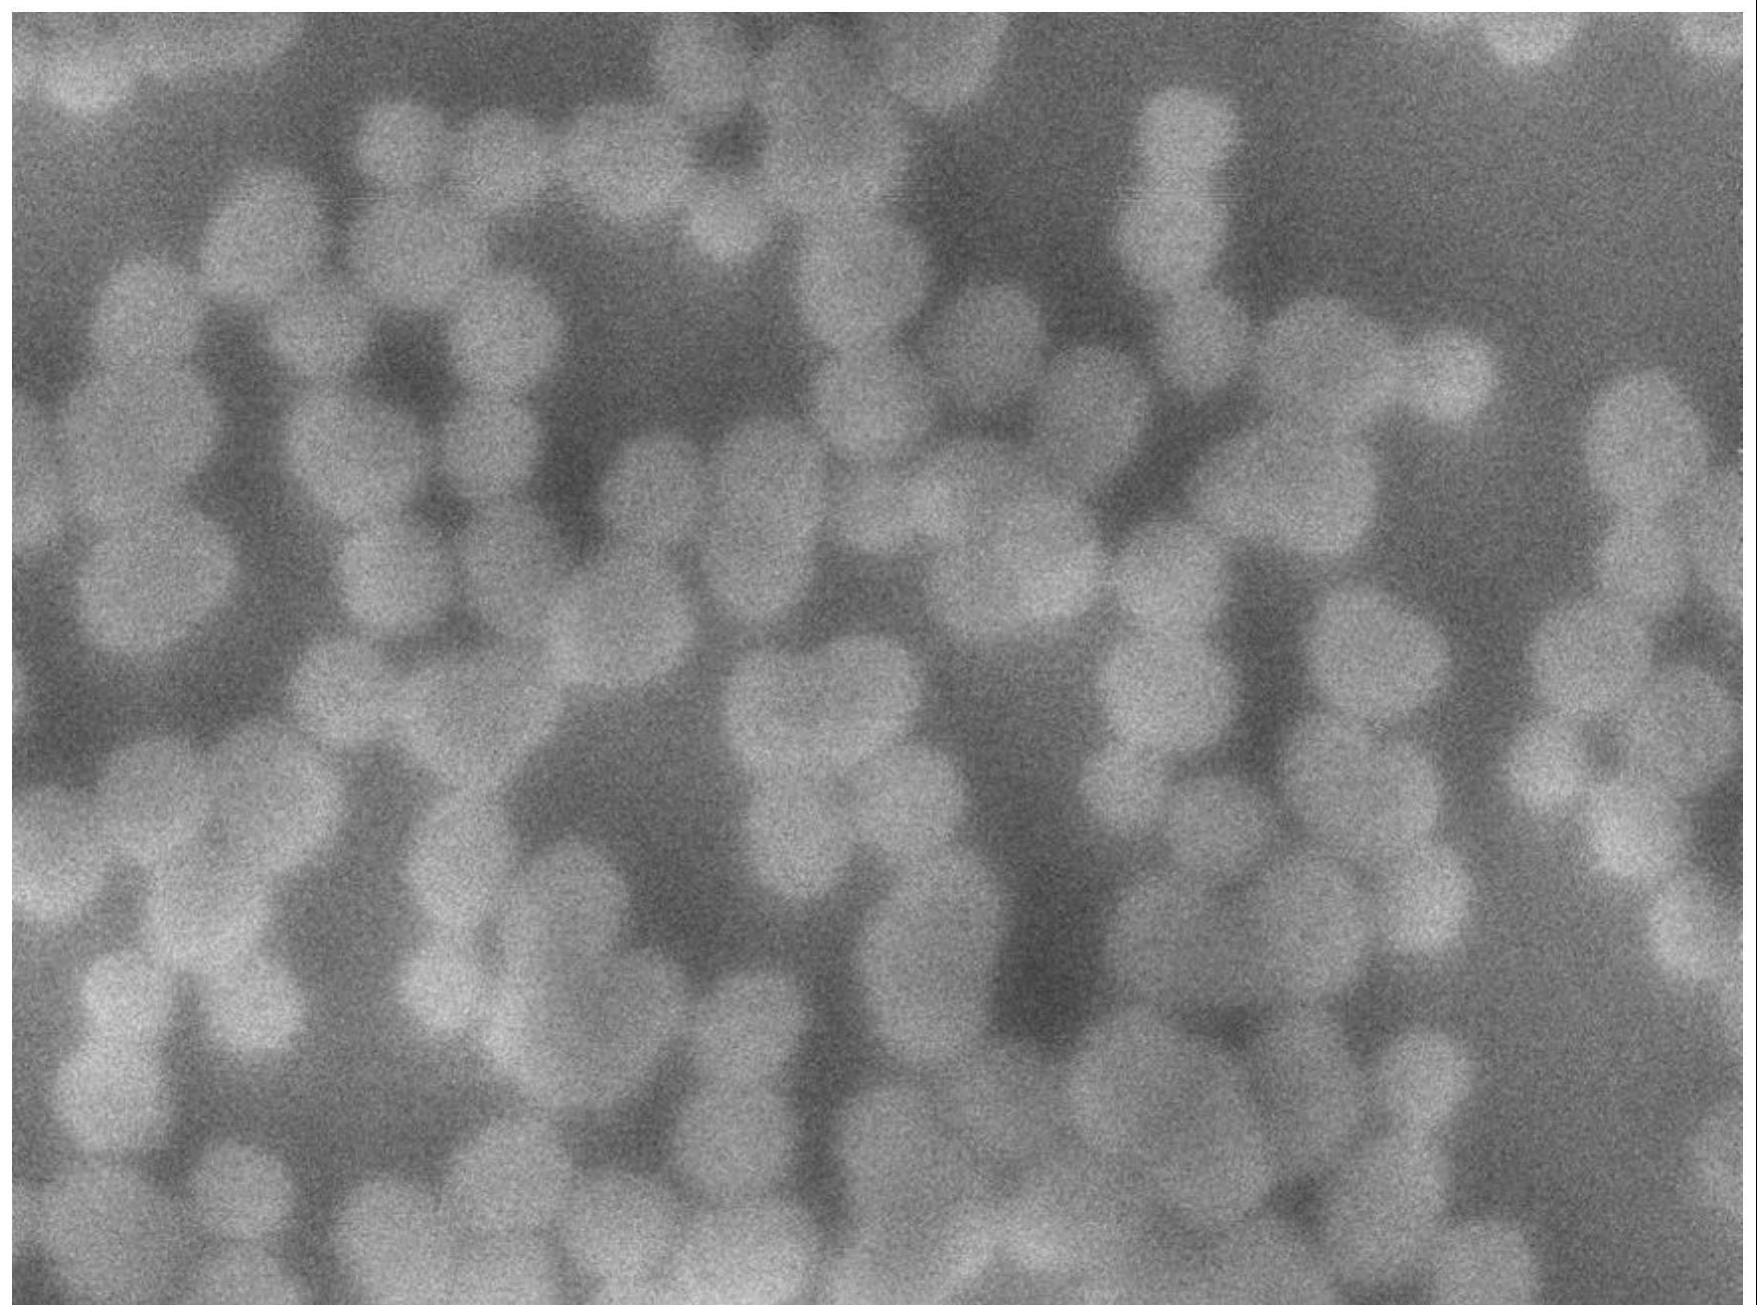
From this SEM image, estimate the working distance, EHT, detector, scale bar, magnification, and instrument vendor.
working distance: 3 mm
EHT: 1 kV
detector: SEI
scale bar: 100 nm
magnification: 200 K X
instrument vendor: JEOL